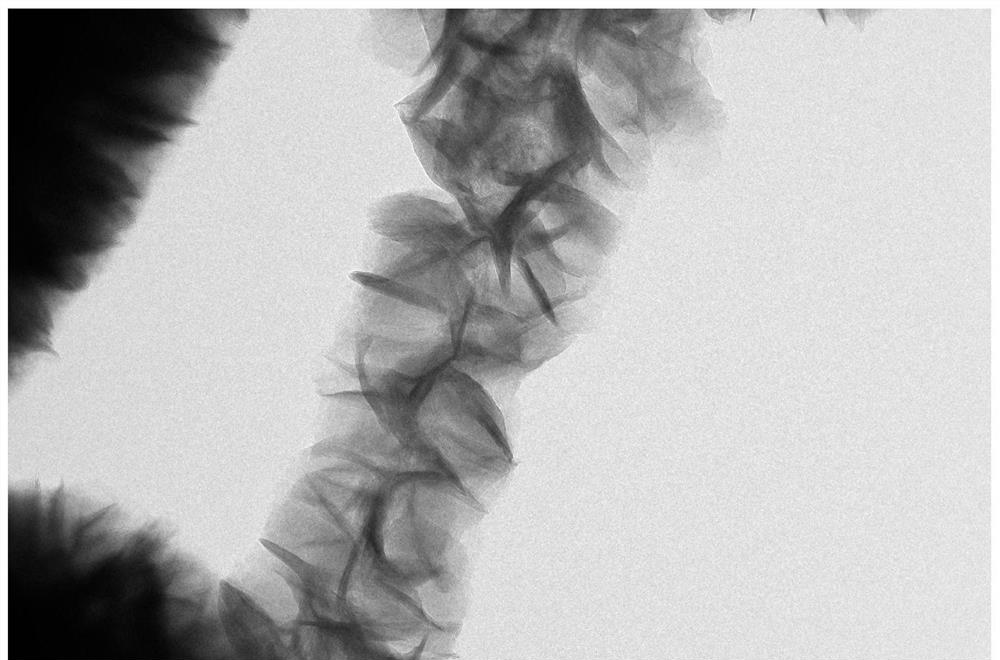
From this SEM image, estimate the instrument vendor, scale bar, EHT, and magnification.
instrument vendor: JEOL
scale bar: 100 nm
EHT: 200 kV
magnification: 80 K X